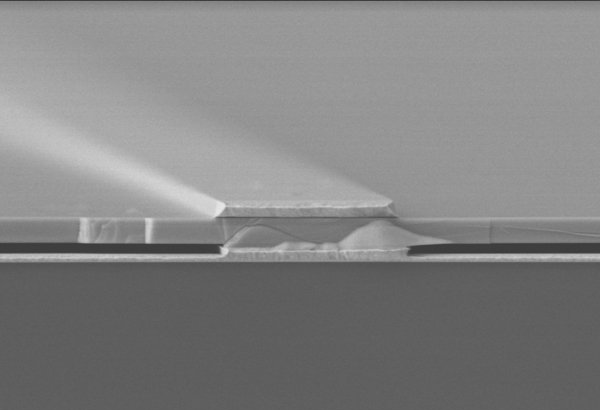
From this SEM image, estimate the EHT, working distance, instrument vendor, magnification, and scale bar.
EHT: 10 kV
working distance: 12.5 mm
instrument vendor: Hitachi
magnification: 10 K X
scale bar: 10000 nm